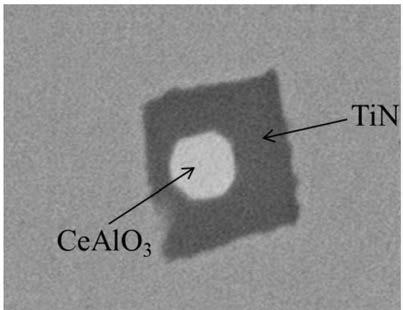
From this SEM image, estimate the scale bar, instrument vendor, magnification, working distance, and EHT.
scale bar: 5000 nm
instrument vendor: other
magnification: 8 K X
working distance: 7.1 mm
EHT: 20 kV